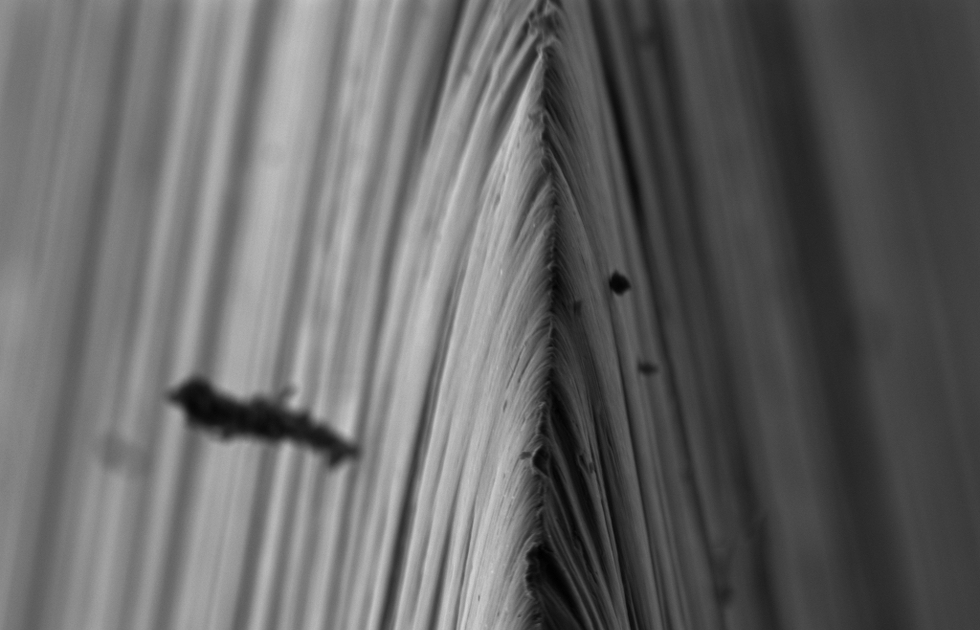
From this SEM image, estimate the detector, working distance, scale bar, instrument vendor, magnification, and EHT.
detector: SE2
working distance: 6.2 mm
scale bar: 1000 nm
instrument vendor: Zeiss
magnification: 10 K X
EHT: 3 kV